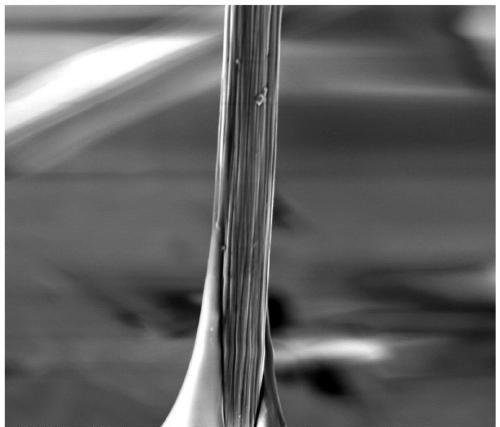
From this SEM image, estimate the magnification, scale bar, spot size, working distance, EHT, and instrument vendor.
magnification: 5 K X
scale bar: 20000 nm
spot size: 4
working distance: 20.8 mm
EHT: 5 kV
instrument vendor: FEI